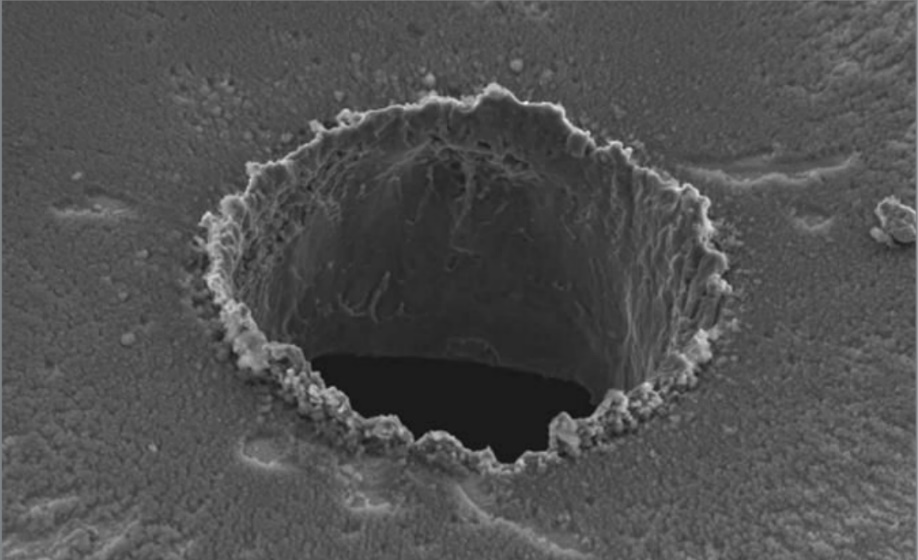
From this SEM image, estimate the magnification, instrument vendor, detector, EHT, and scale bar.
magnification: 0.95 K X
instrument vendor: JEOL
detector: SEI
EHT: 10 kV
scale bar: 20000 nm